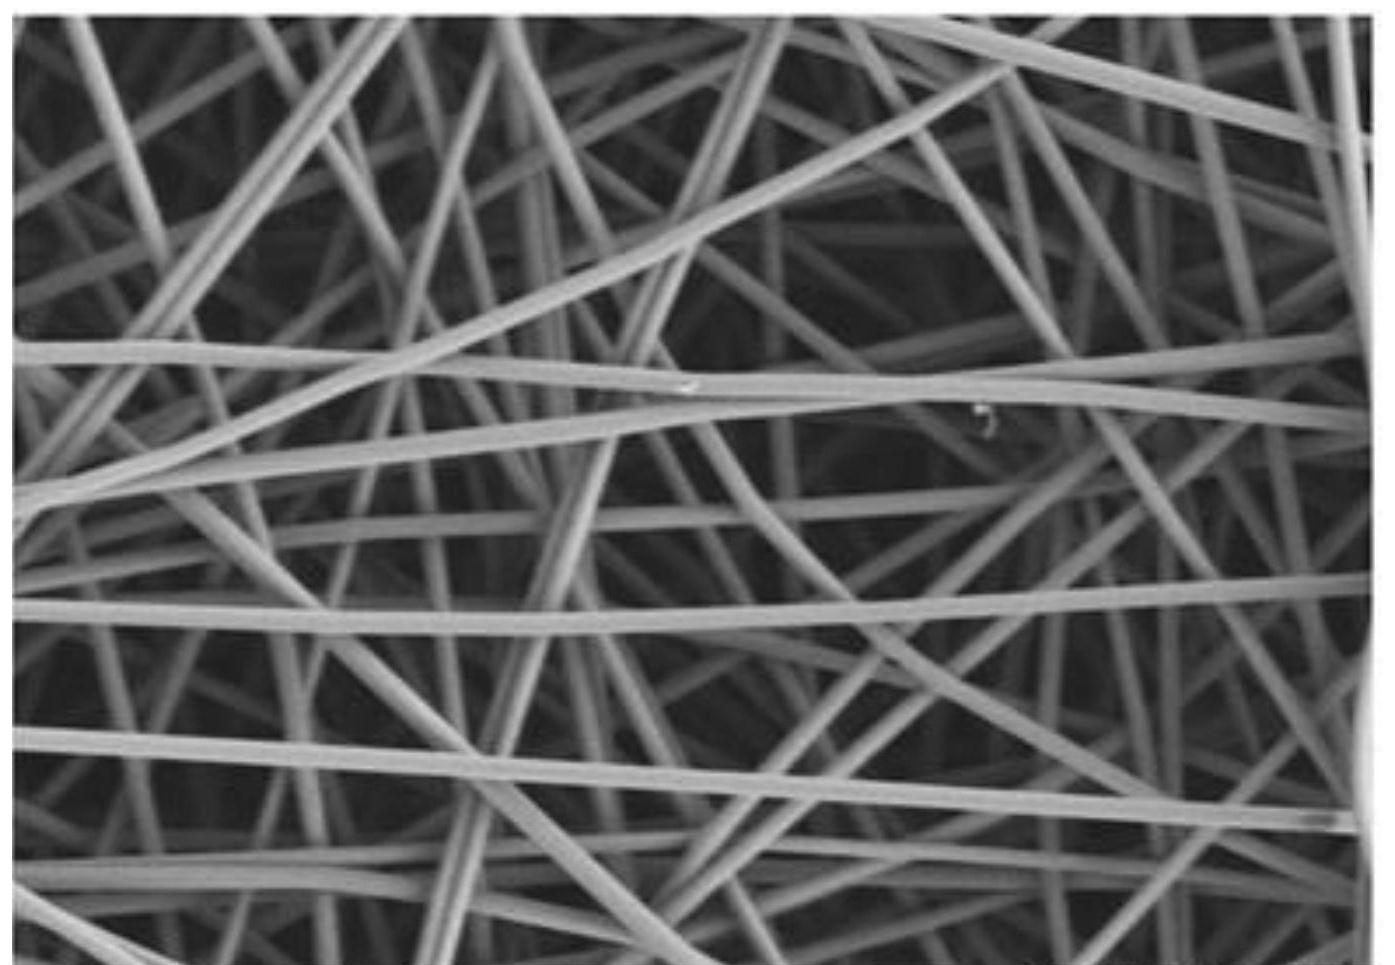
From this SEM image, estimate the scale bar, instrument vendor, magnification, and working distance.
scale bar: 5000 nm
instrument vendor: Hitachi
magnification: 6 K X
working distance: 9.5 mm